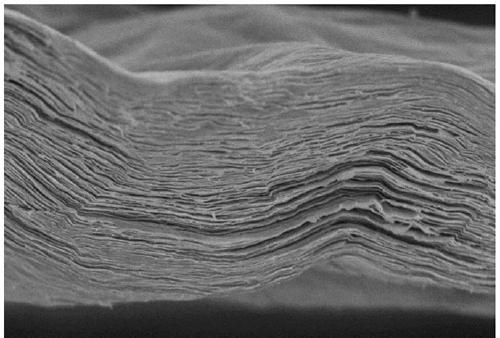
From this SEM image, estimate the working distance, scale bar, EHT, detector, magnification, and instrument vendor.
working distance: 9.7 mm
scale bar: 10000 nm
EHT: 5 kV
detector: SE(M)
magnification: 5 K X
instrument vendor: Hitachi